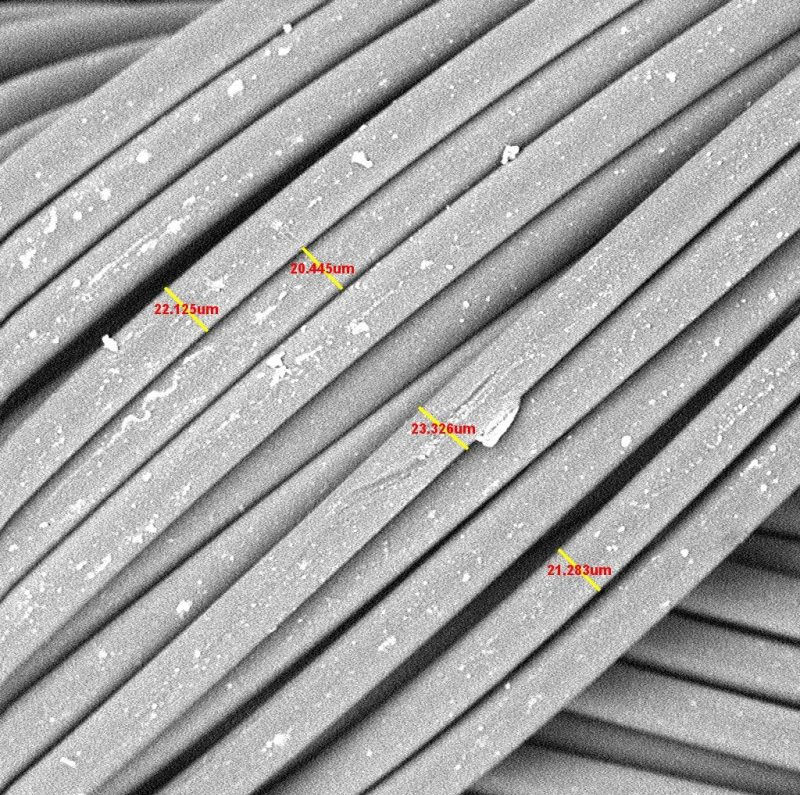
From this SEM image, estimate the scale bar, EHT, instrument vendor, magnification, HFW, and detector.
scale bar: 80000 nm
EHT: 10 kV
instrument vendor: Thermo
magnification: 0.89 K X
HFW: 305 µm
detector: BSD Full